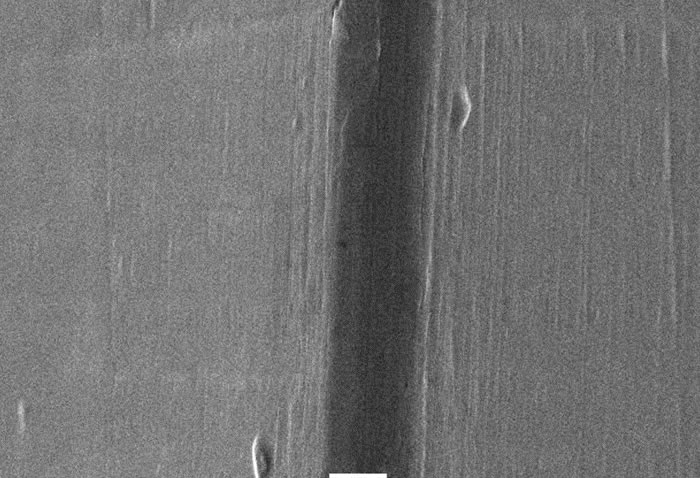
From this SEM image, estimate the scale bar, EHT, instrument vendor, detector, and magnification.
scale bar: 10000 nm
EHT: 20 kV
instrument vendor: JEOL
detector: SEI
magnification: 1 K X